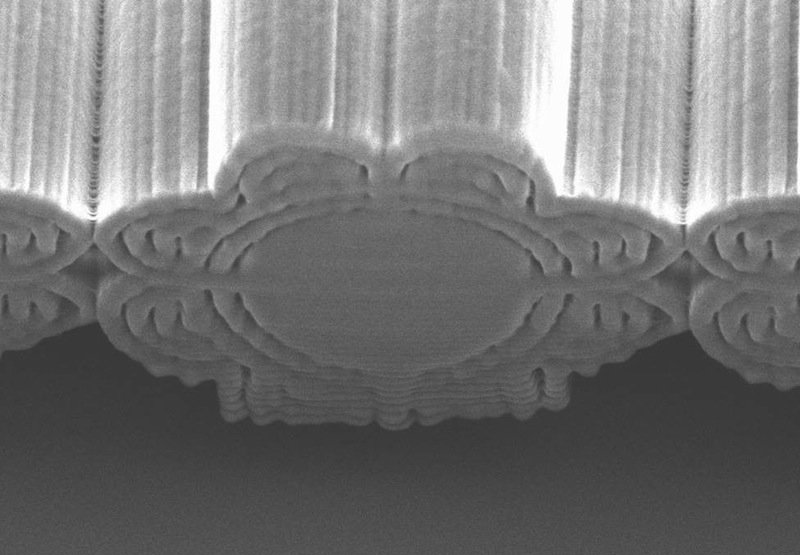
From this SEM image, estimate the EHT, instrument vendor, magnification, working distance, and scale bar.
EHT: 5 kV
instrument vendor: Hitachi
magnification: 6 K X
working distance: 21.6 mm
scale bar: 5000 nm